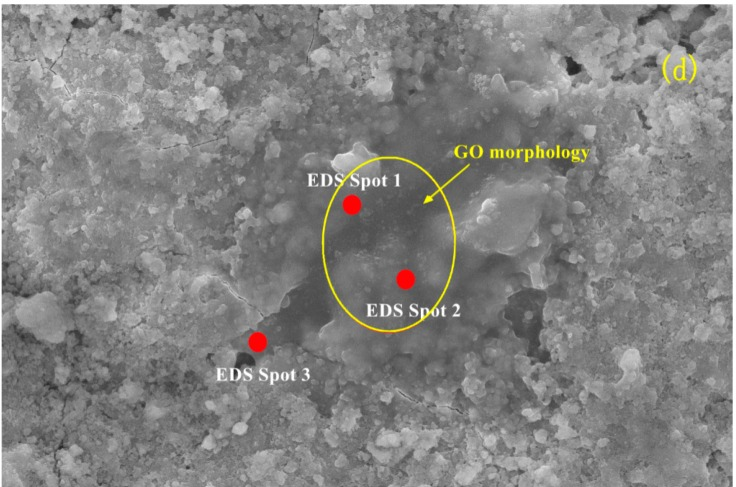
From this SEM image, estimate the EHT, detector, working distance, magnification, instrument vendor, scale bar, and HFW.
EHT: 20 kV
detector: ETD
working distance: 10.1 mm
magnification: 5 K X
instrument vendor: FEI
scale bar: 20000 nm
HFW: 82.9 µm